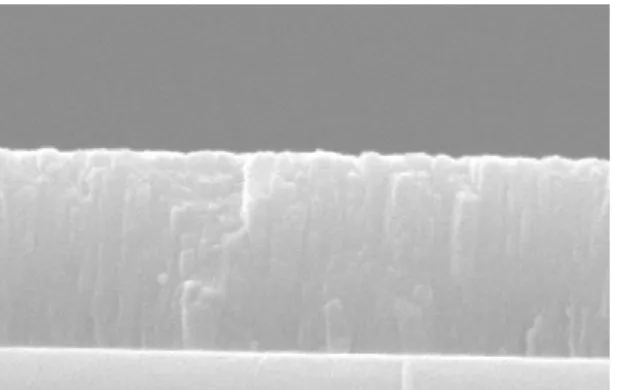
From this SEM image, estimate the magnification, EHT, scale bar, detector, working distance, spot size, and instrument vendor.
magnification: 50 K X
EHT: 15 kV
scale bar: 500 nm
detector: SE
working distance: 10.3 mm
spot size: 3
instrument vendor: FEI